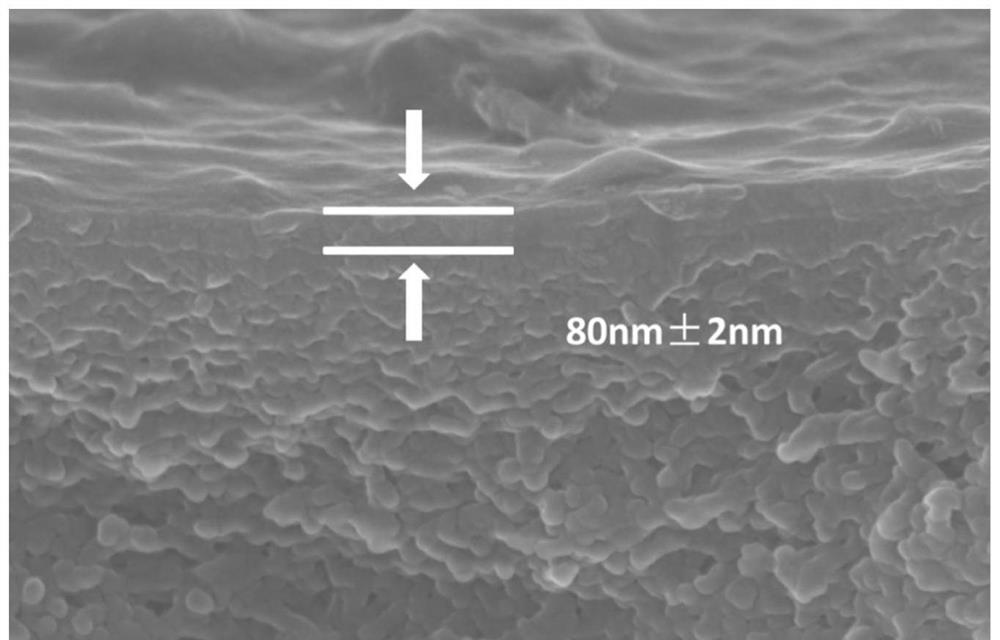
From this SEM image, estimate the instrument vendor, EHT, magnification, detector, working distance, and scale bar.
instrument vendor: Hitachi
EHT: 10 kV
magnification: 50 K X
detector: SE(UL)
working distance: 9.4 mm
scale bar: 1000 nm